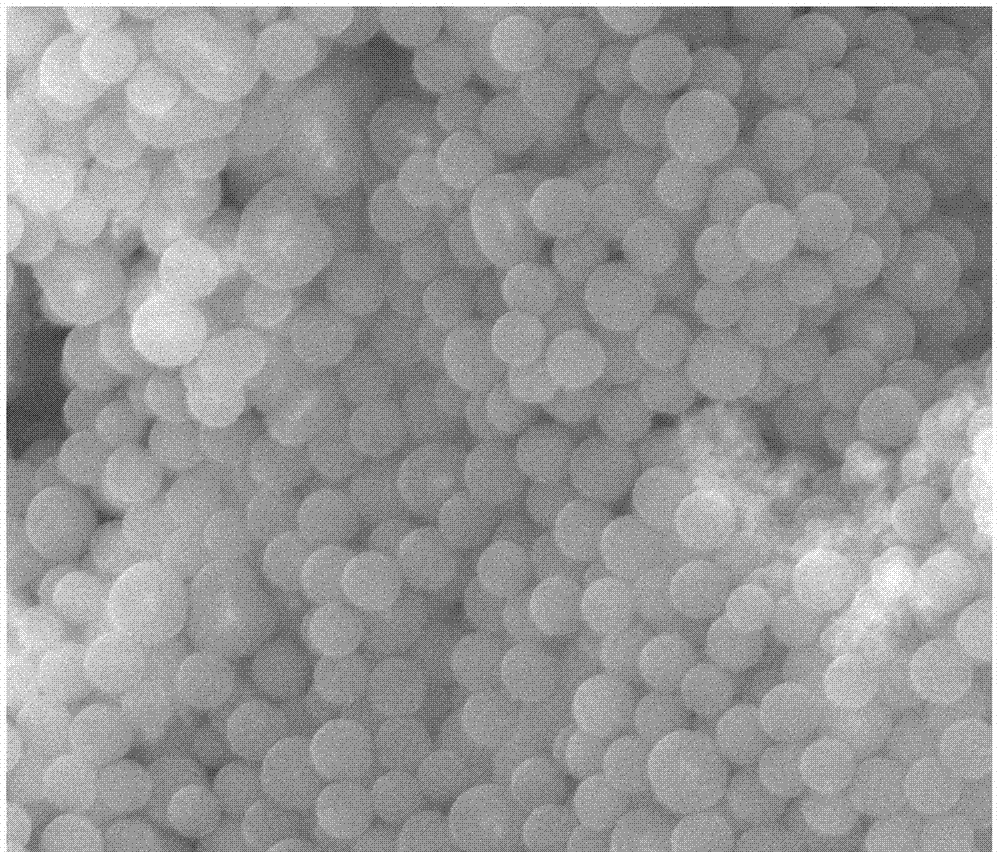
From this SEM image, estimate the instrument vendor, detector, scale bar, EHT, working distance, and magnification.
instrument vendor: FEI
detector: ETD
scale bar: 4000 nm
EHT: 20 kV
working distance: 28 mm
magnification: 40 K X